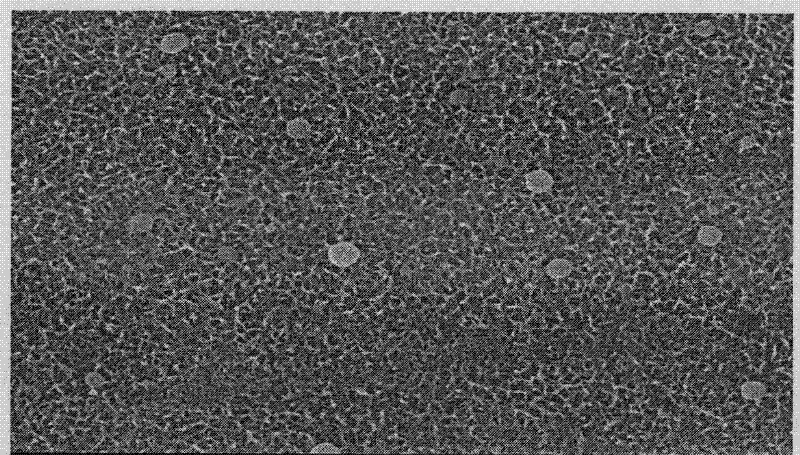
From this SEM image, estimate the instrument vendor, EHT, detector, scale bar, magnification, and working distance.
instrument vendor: Hitachi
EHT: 10 kV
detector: SE(M)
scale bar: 1000 nm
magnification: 30 K X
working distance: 13.1 mm